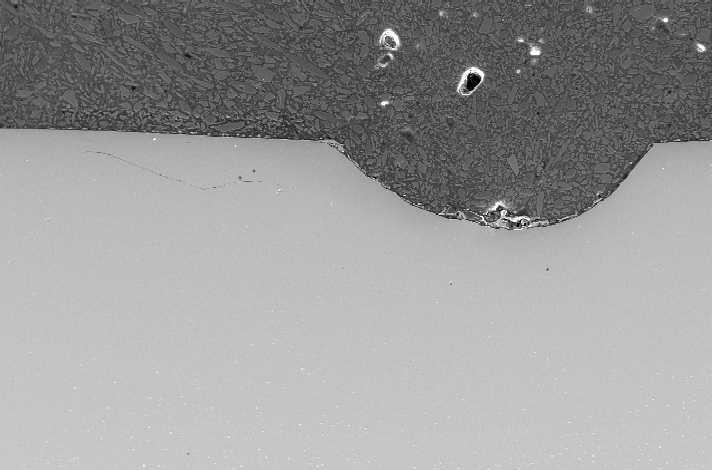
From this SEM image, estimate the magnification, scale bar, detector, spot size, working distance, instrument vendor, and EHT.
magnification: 0.2 K X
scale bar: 200000 nm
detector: SE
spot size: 5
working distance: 10.4 mm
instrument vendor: FEI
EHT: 20 kV